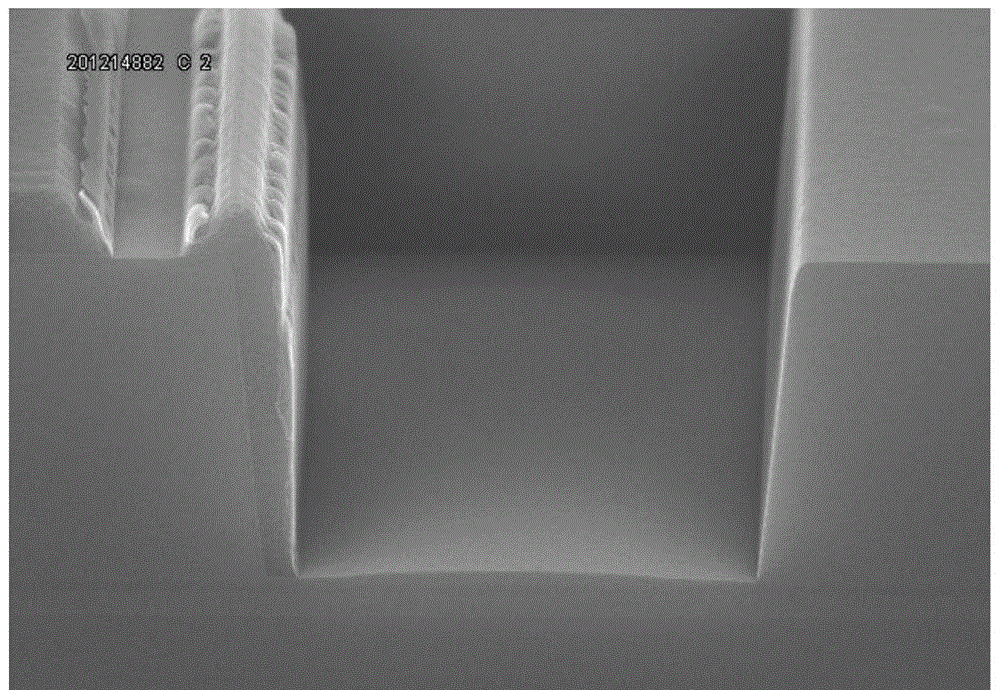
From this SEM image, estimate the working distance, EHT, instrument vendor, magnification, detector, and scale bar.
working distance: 9.3 mm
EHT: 15 kV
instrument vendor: Hitachi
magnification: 15 K X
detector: SE(U)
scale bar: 3000 nm